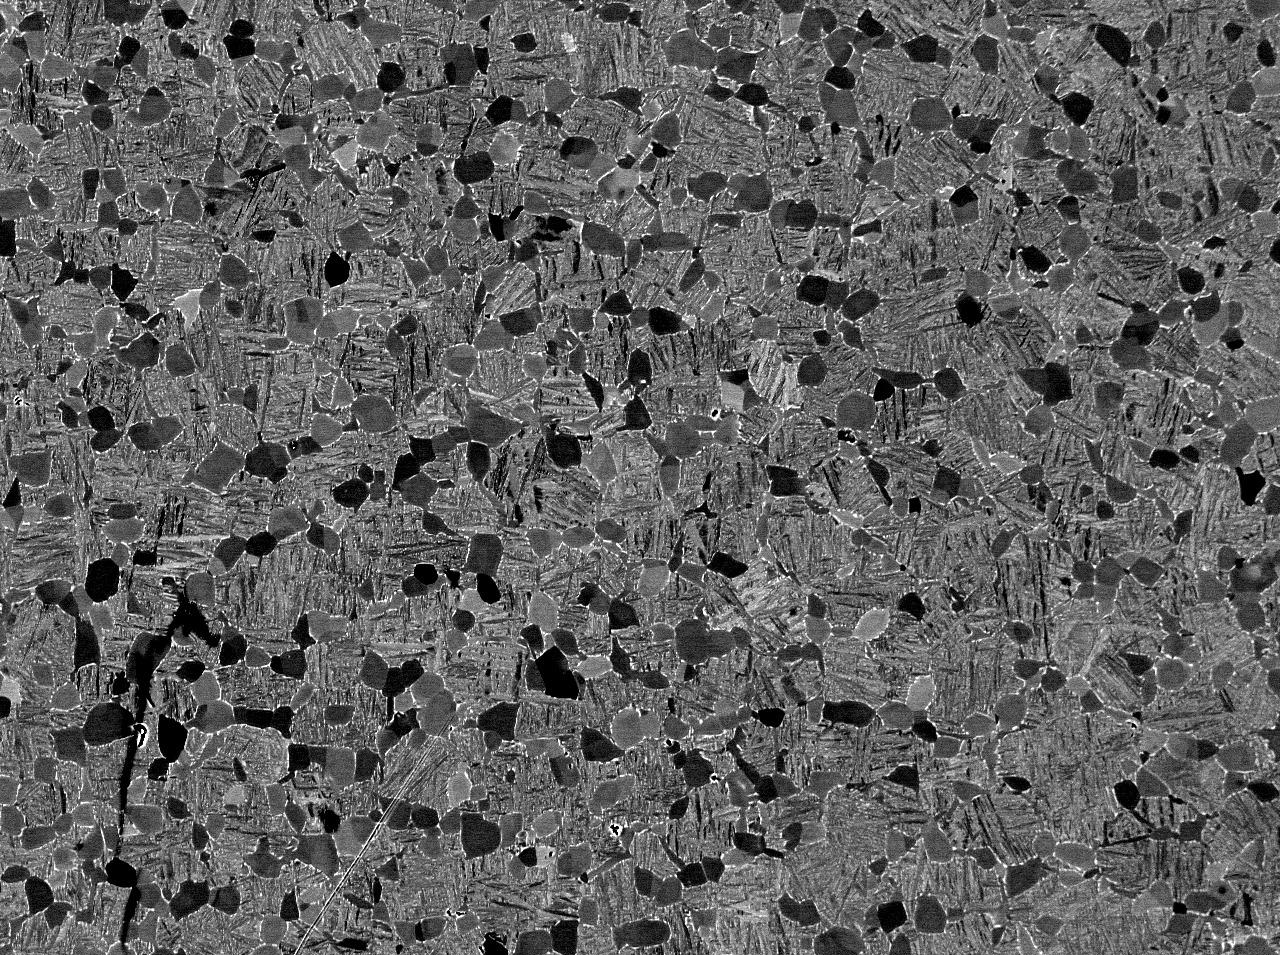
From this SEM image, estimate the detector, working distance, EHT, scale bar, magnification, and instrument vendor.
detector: COMPO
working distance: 10 mm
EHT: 15 kV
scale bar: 10000 nm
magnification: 0.5 K X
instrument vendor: JEOL